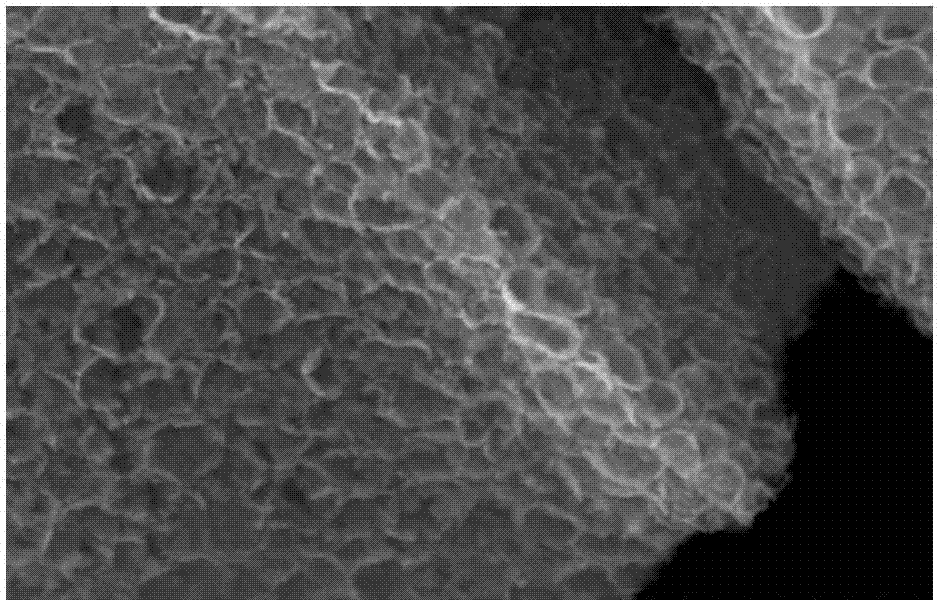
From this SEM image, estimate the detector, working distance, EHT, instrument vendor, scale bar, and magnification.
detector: SE(M)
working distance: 9.7 mm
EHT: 15 kV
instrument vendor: Hitachi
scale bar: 3000 nm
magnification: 15 K X